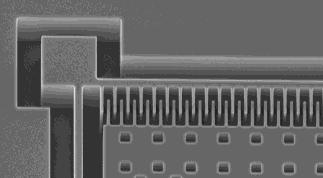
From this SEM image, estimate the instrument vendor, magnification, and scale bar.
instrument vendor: JEOL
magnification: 0.37 K X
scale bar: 50000 nm